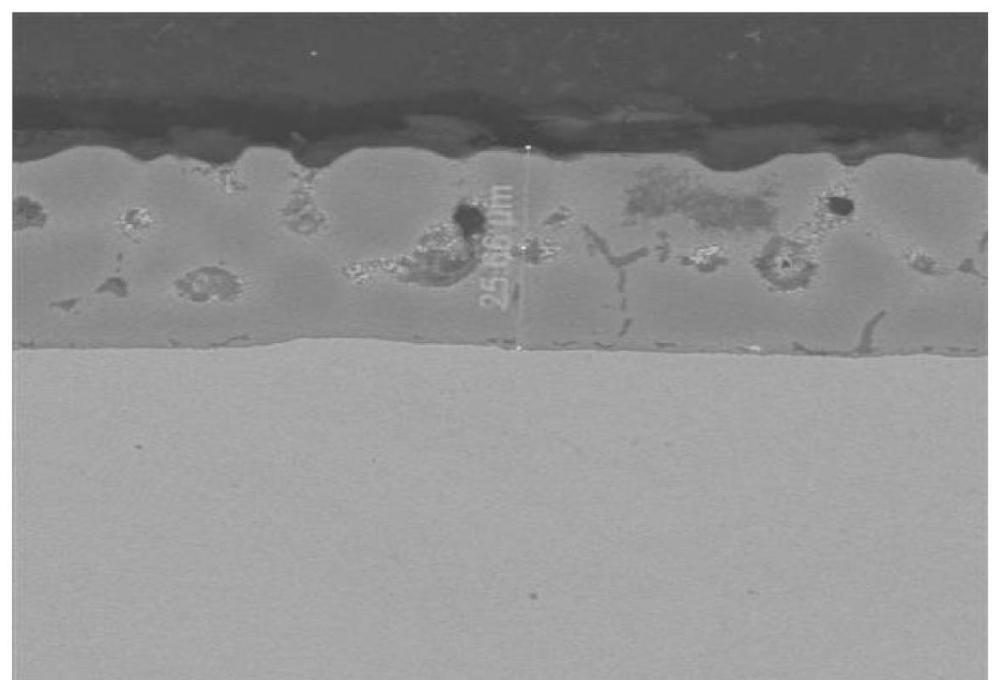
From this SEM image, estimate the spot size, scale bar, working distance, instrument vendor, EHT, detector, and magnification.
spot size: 3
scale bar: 40000 nm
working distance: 9.9 mm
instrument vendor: FEI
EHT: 15 kV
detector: vCD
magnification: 1.5 K X